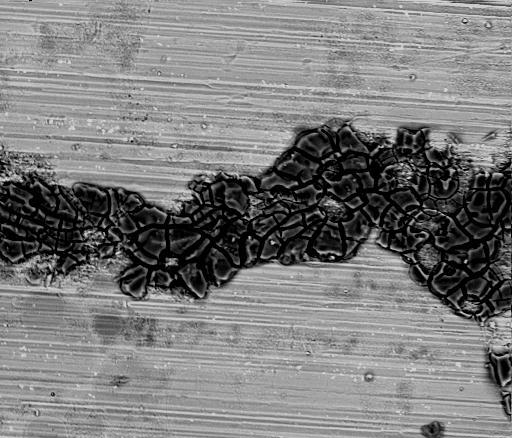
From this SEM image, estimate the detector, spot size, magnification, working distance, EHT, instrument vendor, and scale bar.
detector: SSD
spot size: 5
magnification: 0.2 K X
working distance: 12 mm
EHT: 25 kV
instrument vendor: FEI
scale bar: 300000 nm